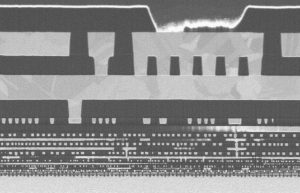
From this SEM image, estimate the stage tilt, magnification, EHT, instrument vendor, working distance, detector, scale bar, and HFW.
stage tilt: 52°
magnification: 50 K X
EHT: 1.3 kV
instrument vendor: FEI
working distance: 4 mm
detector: TLD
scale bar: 2000 nm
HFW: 8.29 µm